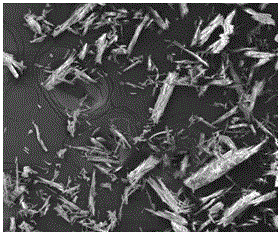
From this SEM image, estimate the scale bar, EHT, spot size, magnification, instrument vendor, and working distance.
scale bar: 300000 nm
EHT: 15 kV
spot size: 6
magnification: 0.42 K X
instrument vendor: FEI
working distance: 11.3 mm